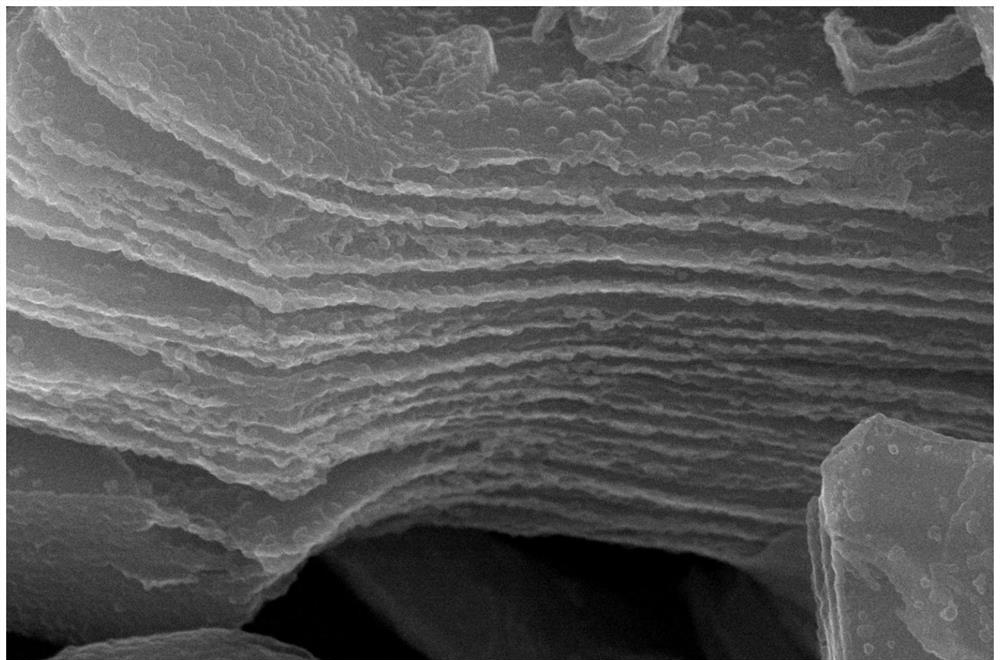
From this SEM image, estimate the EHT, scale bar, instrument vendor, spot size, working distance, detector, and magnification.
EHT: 20 kV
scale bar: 1000 nm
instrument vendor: FEI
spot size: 3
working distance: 6.8 mm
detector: SE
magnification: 100 K X